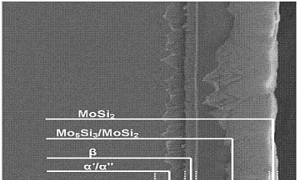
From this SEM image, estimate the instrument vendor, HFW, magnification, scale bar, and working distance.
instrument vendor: FEI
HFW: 56.33 µm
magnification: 2.4 K X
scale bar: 20000 nm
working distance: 9.5 mm